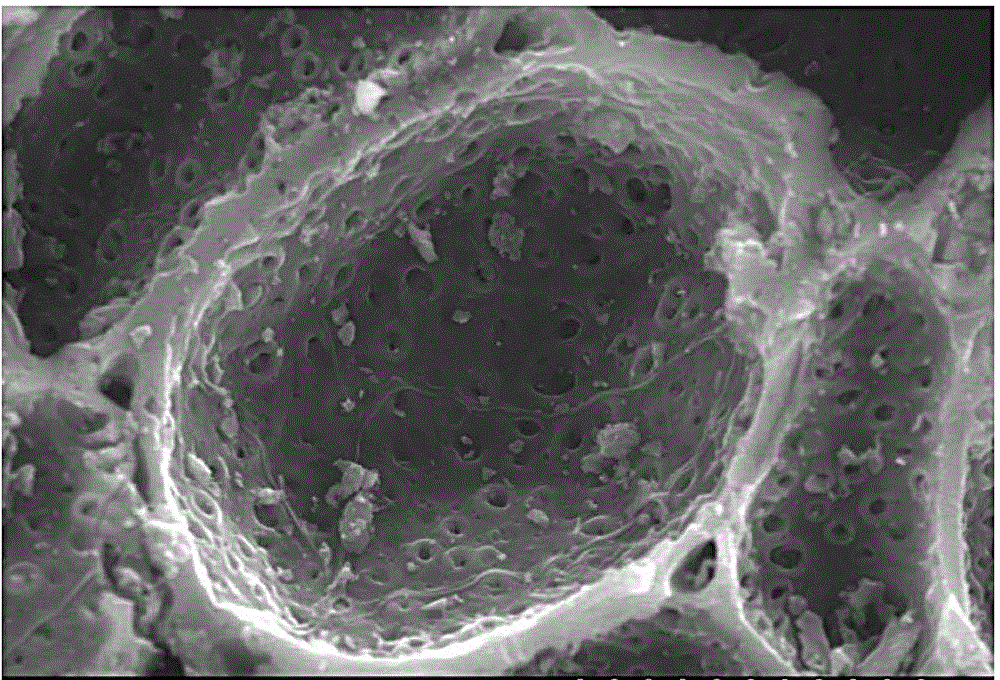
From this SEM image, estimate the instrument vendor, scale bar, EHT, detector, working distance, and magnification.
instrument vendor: Hitachi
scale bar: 25000 nm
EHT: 15 kV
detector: SE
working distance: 10.7 mm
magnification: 1.8 K X